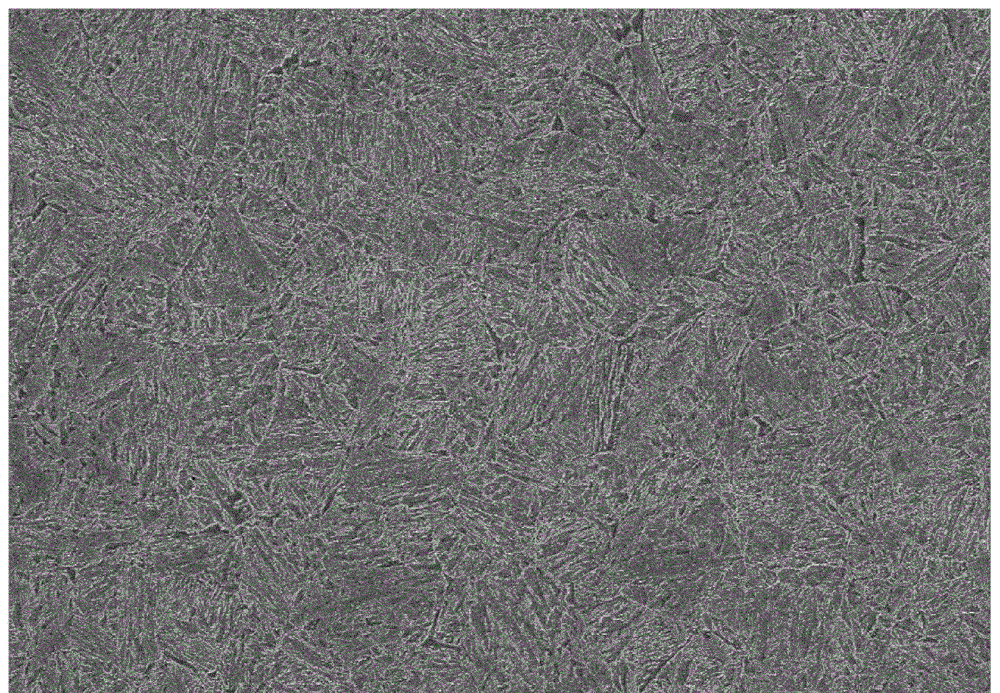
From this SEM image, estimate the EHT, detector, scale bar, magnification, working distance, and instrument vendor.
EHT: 25 kV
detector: SE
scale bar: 50000 nm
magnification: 1 K X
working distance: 6.3 mm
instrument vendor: Hitachi